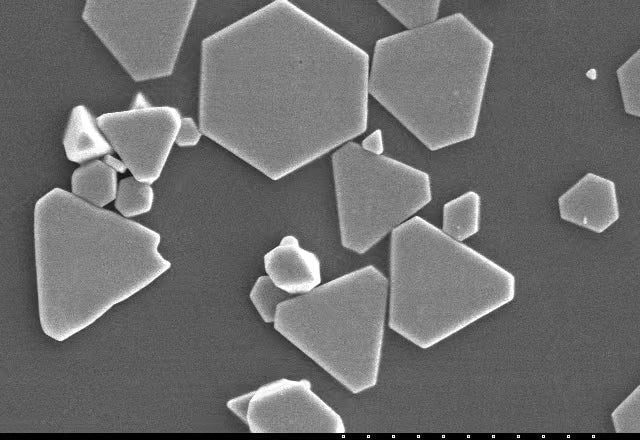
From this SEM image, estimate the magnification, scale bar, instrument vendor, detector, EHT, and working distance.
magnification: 5 K X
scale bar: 10000 nm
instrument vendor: Hitachi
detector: SE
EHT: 10 kV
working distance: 12.7 mm